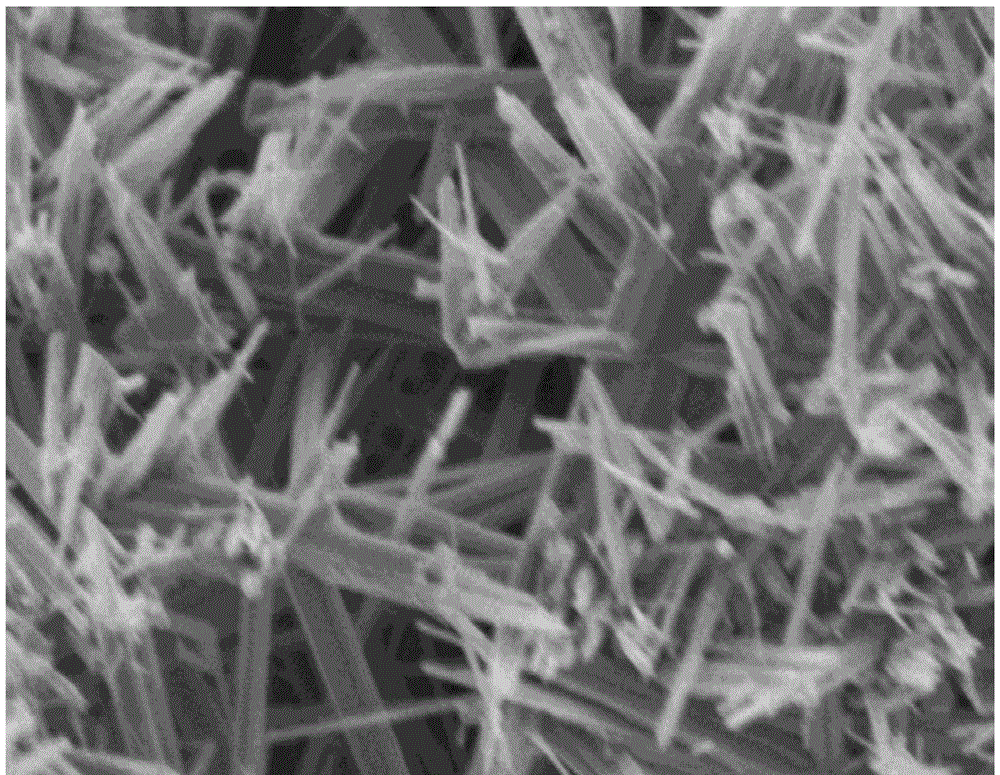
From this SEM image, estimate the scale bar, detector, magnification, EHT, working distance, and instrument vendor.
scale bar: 500 nm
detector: SE(M)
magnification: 60 K X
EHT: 2 kV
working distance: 5 mm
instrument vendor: Hitachi